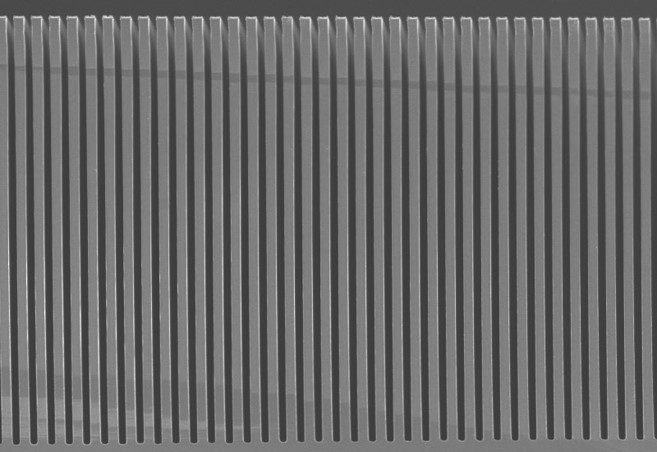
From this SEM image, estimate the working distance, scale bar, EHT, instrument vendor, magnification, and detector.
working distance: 7.7 mm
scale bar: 100000 nm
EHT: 5 kV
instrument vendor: Hitachi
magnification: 0.5 K X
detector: SE(UL)